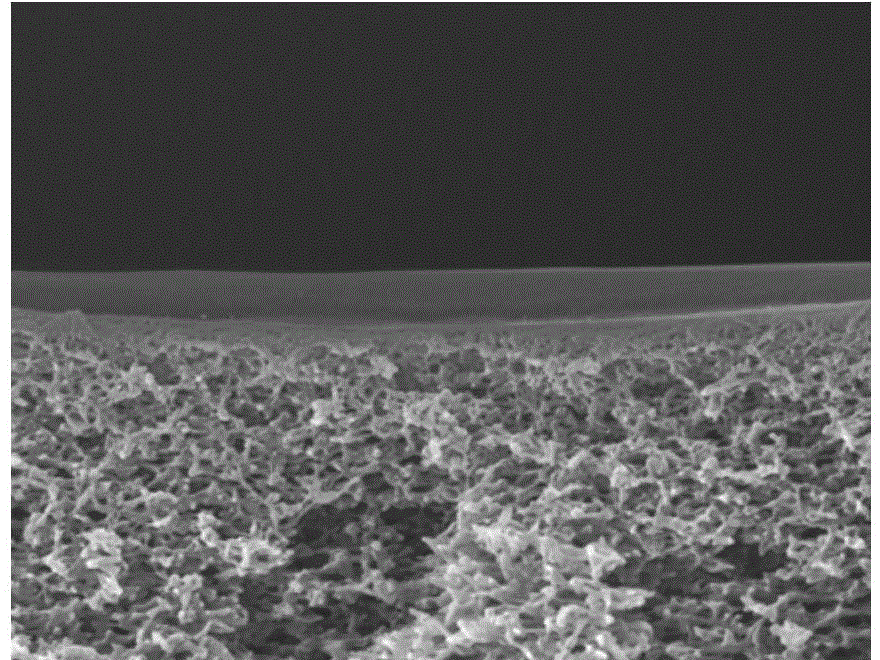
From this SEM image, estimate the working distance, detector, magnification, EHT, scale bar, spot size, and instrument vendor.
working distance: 4.4 mm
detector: TLD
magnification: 50 K X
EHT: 10 kV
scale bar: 1000 nm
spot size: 3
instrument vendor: FEI